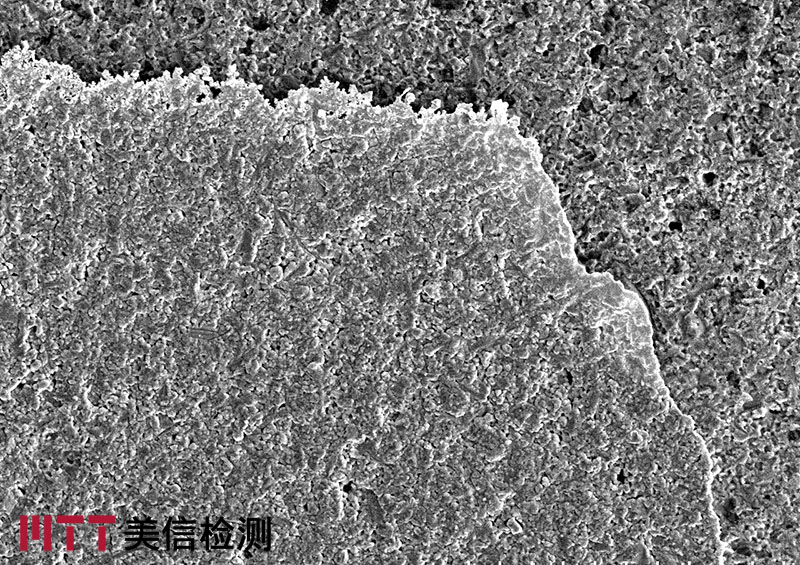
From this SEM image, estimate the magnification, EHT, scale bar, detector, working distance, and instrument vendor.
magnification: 1 K X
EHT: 15 kV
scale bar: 50000 nm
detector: SE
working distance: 10 mm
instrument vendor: Hitachi